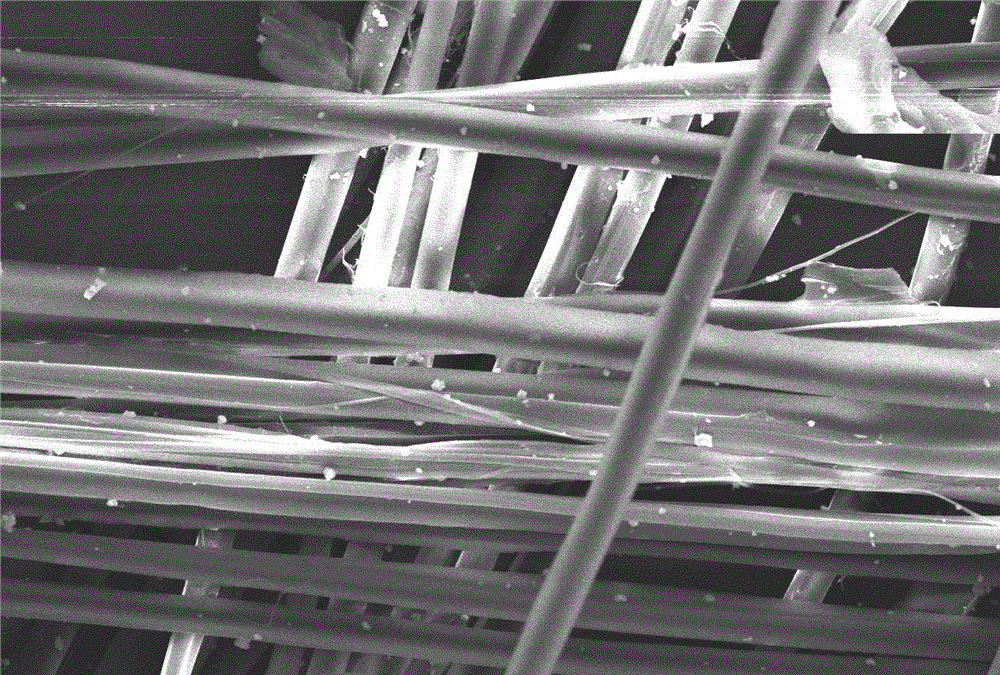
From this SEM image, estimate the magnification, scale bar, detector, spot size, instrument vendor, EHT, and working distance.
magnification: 0.5 K X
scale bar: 50000 nm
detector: SEI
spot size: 40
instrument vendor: JEOL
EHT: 20 kV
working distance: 8 mm